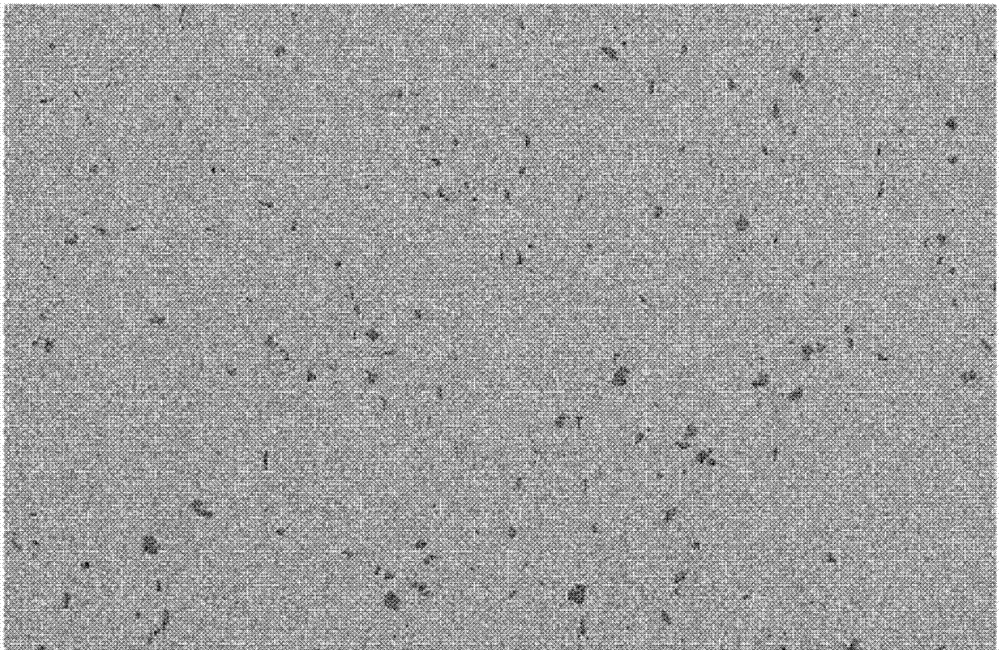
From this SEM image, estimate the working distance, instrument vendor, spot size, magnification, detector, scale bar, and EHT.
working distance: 6.4 mm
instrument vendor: FEI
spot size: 3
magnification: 1 K X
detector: SE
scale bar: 50000 nm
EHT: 20 kV